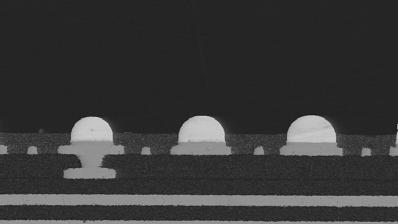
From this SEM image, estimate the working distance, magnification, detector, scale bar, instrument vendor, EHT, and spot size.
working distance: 5 mm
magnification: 0.15 K X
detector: BSE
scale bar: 100000 nm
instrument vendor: FEI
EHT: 15 kV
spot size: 5